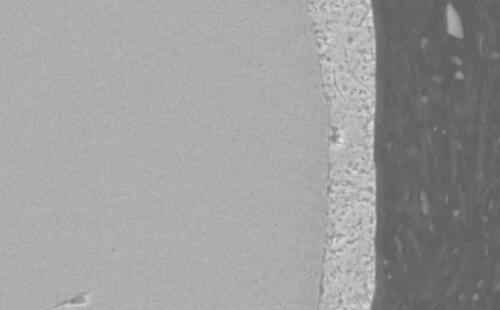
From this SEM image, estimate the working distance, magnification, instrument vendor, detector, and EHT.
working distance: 10.1 mm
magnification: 1.1 K X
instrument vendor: JEOL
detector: SED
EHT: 20 kV